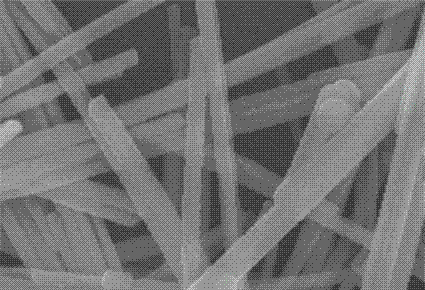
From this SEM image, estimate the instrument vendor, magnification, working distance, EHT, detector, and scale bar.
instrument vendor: Hitachi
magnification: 80 K X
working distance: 9.4 mm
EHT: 10 kV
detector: SE(M,LA0)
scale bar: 500 nm